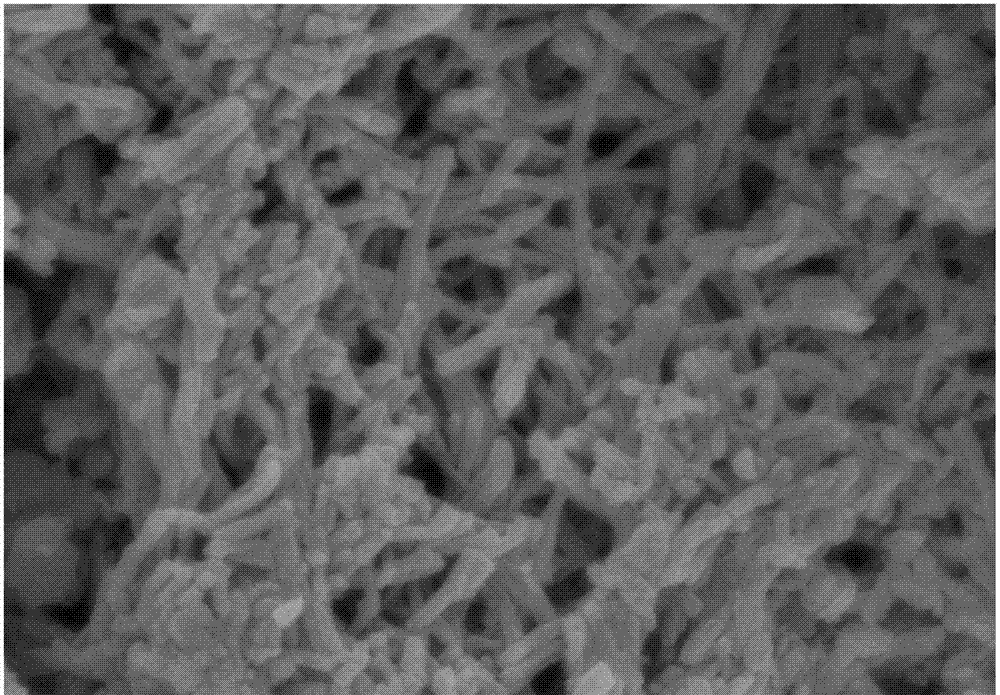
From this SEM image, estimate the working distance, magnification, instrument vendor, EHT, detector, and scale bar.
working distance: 9 mm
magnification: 40 K X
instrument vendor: Hitachi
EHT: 3 kV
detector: SE(M)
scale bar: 1000 nm